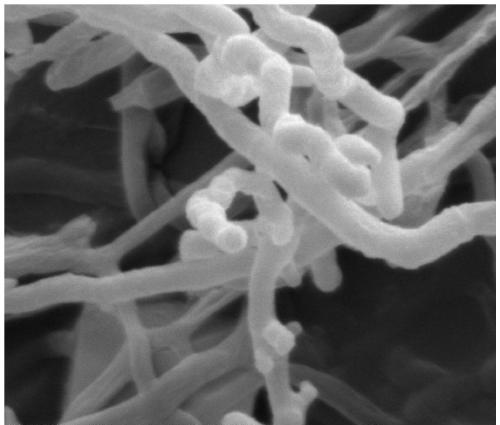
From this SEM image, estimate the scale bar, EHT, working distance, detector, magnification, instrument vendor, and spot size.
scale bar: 2000 nm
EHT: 15 kV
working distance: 10.3 mm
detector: ETD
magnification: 15 K X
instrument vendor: FEI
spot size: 3